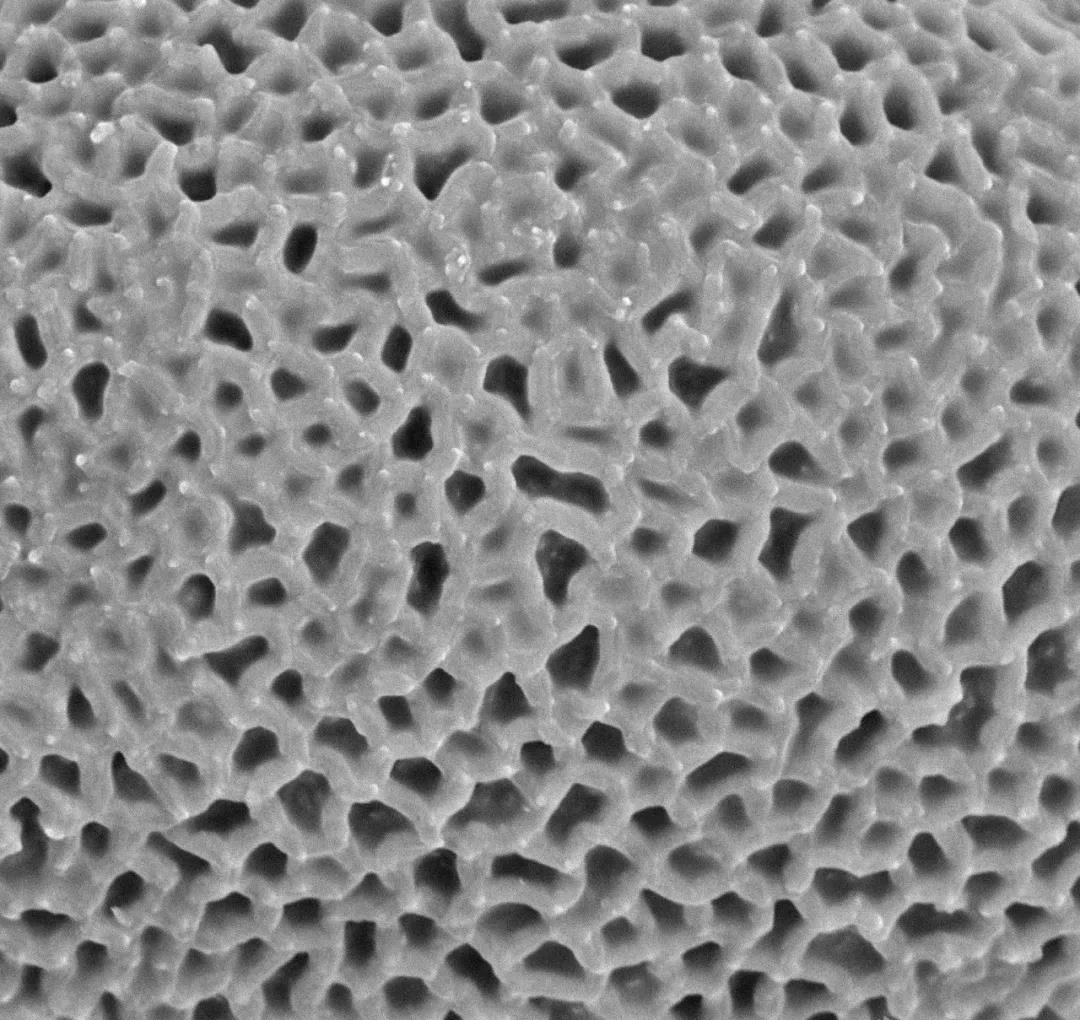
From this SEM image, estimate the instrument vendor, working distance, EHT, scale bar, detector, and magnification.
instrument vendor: other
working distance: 5.4 mm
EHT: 15 kV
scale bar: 5000 nm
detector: BSE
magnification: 10 K X